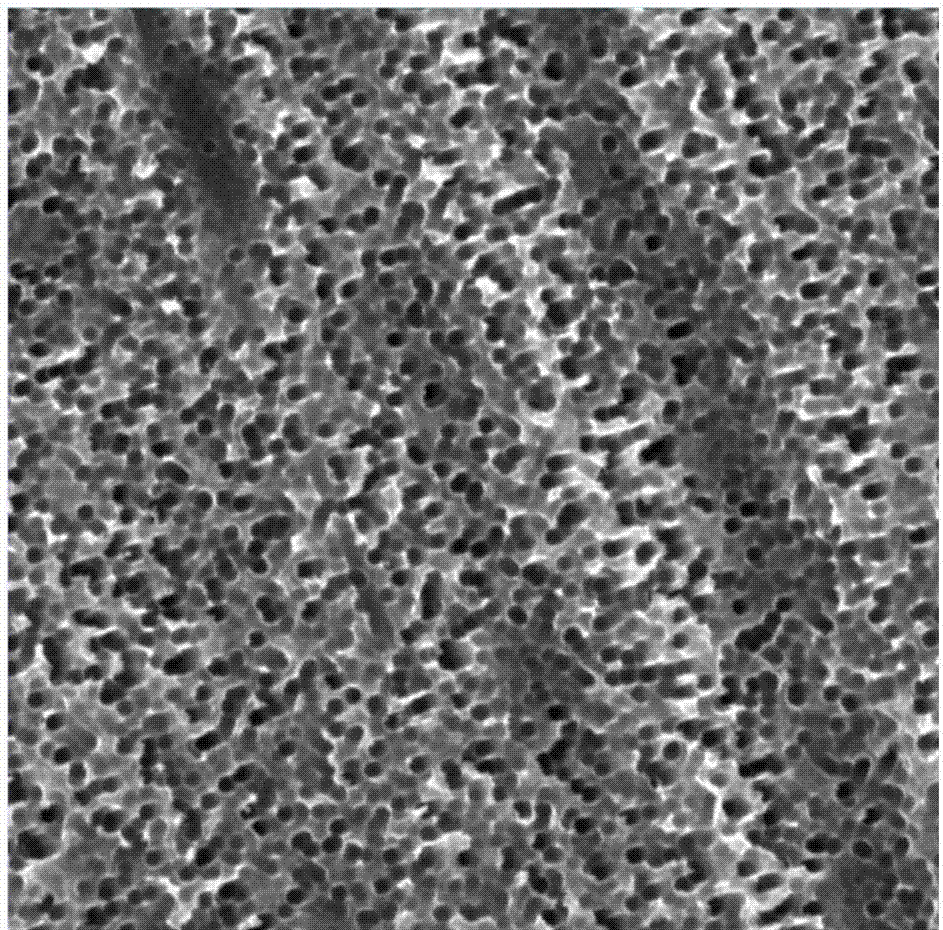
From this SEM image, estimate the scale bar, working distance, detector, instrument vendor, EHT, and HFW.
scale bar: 5000 nm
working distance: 15.63 mm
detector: SE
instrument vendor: Tescan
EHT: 20 kV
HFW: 30.96 µm